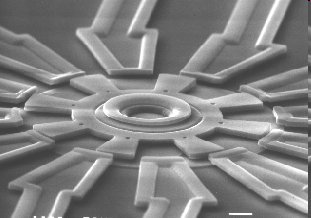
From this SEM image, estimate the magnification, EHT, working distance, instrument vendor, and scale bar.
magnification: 0.85 K X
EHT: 30 kV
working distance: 25 mm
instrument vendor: JEOL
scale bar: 10000 nm